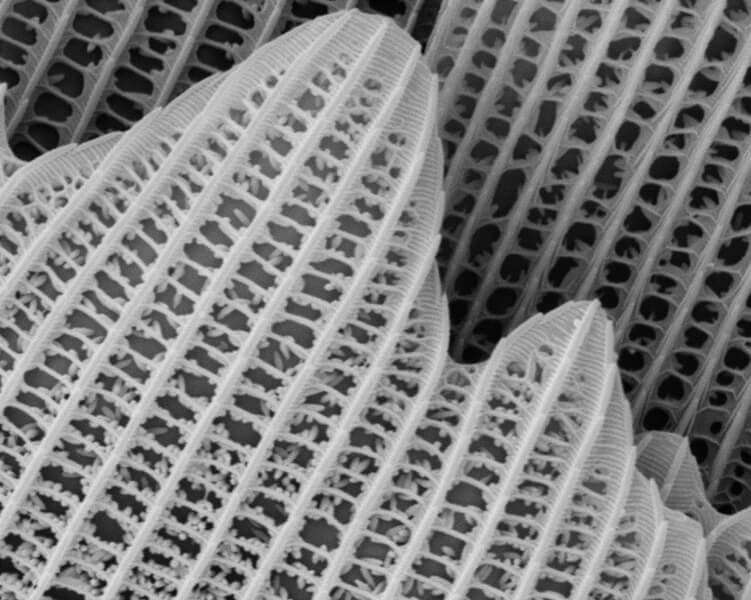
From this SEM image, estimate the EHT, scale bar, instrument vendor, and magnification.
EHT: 10 kV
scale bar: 5000 nm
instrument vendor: JEOL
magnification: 5 K X